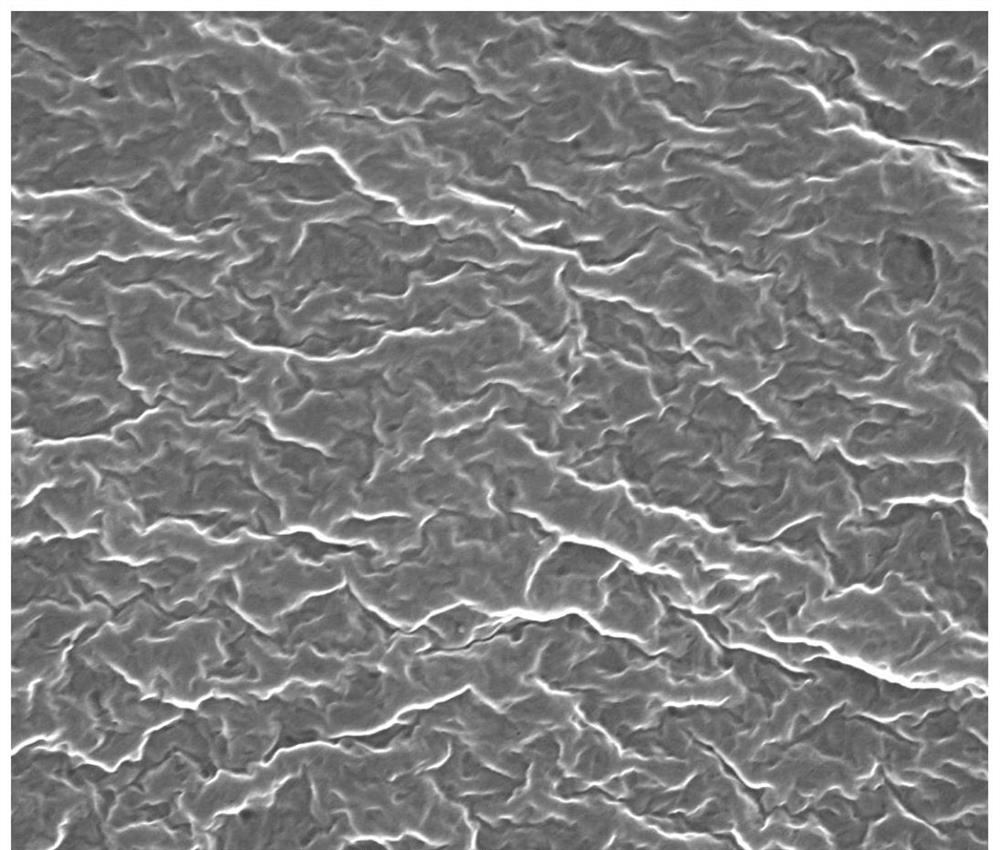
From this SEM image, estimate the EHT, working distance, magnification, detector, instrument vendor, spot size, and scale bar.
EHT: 15 kV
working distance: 4.8 mm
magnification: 80 K X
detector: TLD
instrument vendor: FEI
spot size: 3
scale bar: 1000 nm